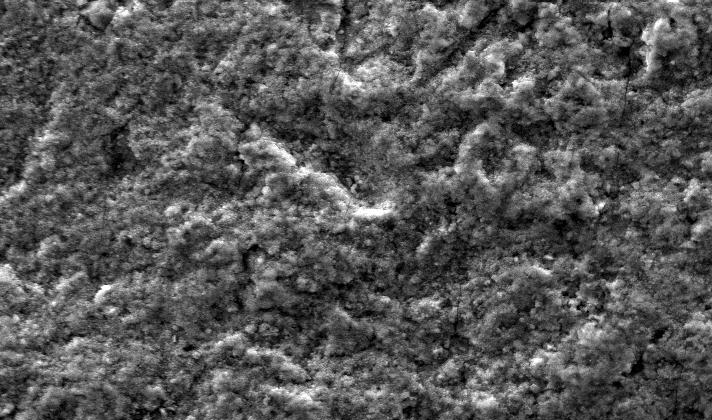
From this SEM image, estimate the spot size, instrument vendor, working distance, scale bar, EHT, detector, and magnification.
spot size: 3.6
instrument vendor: FEI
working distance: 6.8 mm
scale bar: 20000 nm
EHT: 25 kV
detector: SE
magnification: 0.8 K X